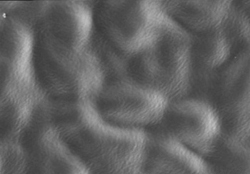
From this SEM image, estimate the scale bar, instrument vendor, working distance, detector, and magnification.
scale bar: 30000 nm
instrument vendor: Hitachi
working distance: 14.7 mm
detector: SE(U)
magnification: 1.8 K X